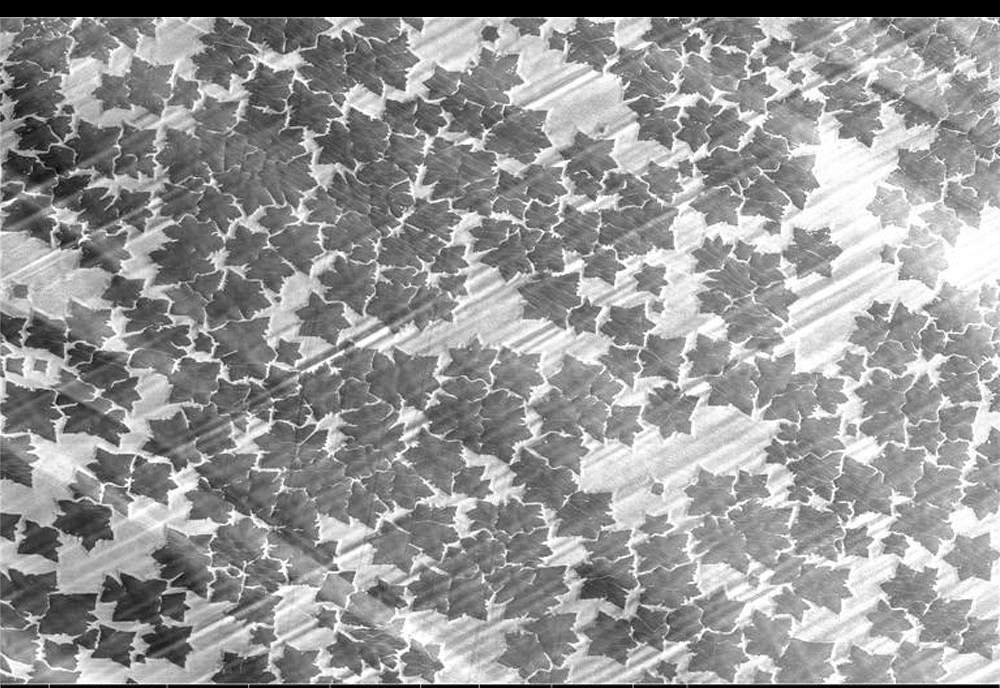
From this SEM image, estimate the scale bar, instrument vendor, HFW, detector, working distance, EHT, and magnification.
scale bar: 300000 nm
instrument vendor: FEI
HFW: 1380 µm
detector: ETD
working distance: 4 mm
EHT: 5 kV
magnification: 0.3 K X